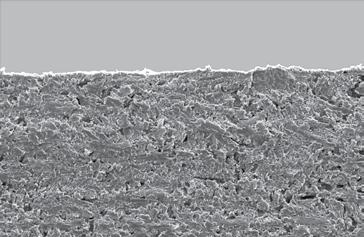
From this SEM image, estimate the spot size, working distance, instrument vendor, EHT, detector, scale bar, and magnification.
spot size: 5.2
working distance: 12.2 mm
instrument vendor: FEI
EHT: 16 kV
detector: SE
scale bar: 200000 nm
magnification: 0.1 K X